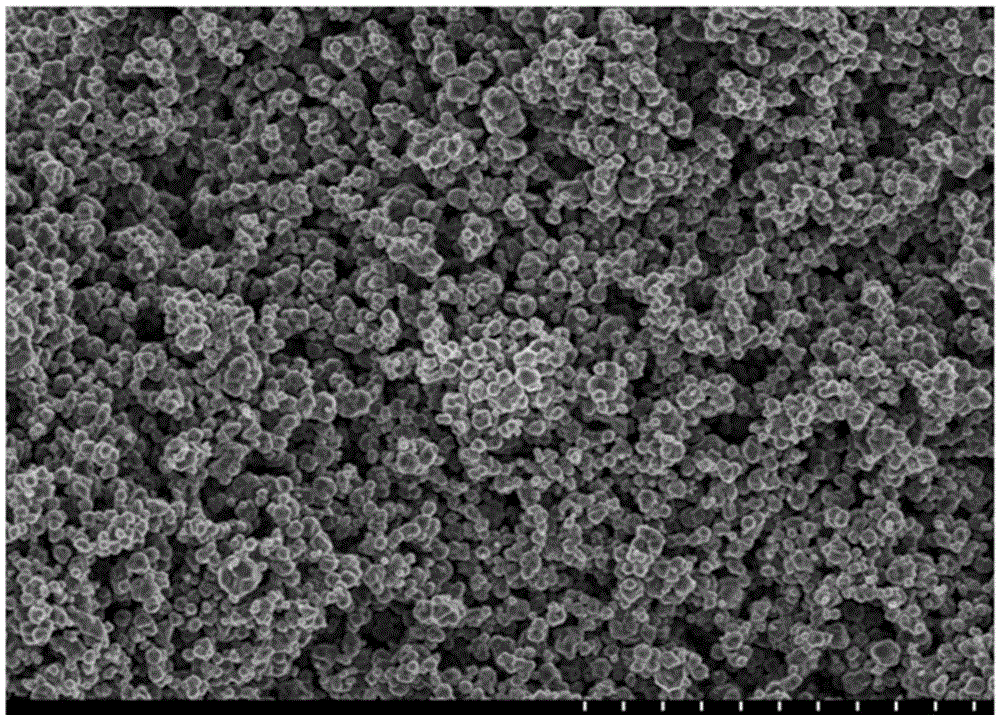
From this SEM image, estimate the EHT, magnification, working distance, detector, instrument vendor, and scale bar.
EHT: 3 kV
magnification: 5 K X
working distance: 7.9 mm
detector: SE(M)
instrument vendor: Hitachi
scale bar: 100000 nm